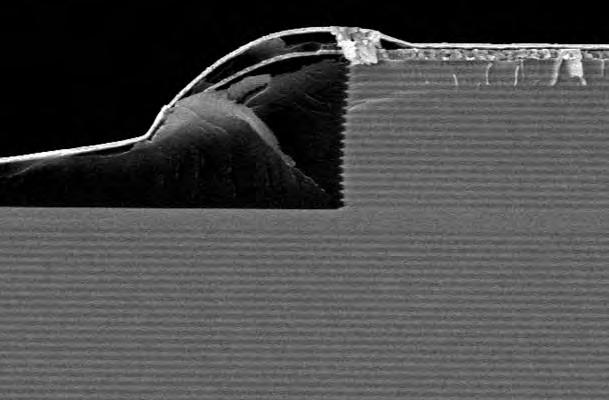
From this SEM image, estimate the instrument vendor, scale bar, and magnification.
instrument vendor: Hitachi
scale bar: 6000 nm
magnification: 5 K X